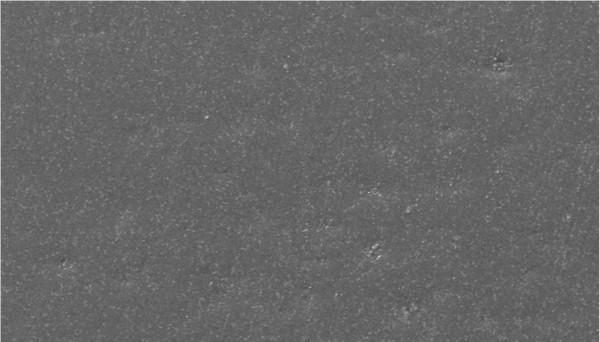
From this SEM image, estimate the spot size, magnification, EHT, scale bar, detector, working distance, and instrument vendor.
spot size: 4.8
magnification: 0.5 K X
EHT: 10 kV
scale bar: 50000 nm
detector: SE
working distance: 10 mm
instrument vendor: FEI